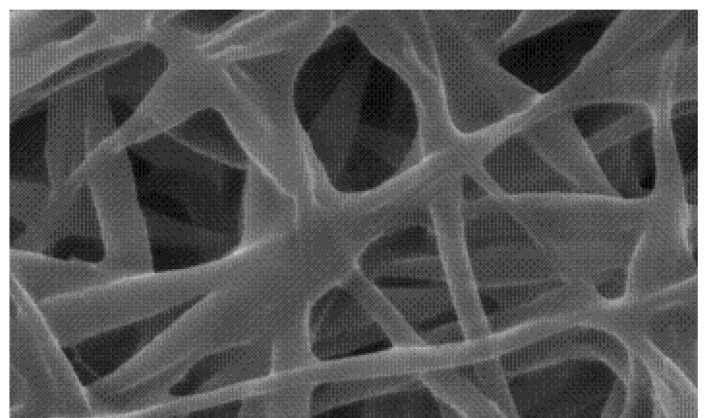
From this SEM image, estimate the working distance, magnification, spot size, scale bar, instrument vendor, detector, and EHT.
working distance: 6.6 mm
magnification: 20 K X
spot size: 4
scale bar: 2000 nm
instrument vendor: FEI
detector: SE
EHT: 25 kV